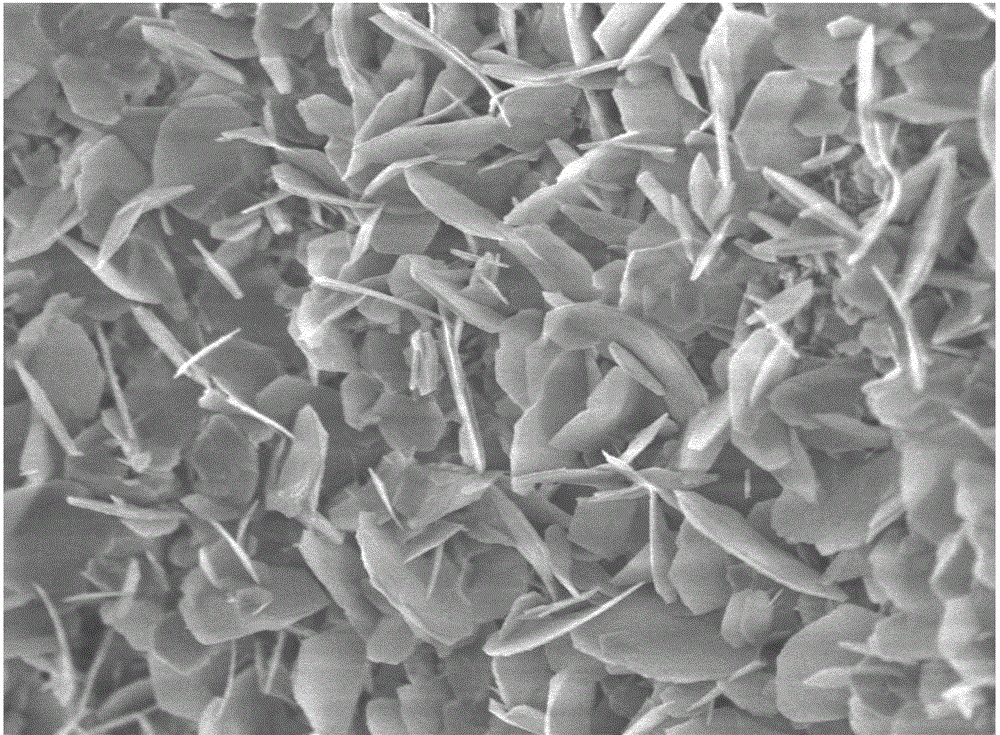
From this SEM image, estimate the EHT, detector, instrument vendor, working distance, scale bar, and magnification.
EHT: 3 kV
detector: UED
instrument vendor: JEOL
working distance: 3 mm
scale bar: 1000 nm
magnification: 10 K X